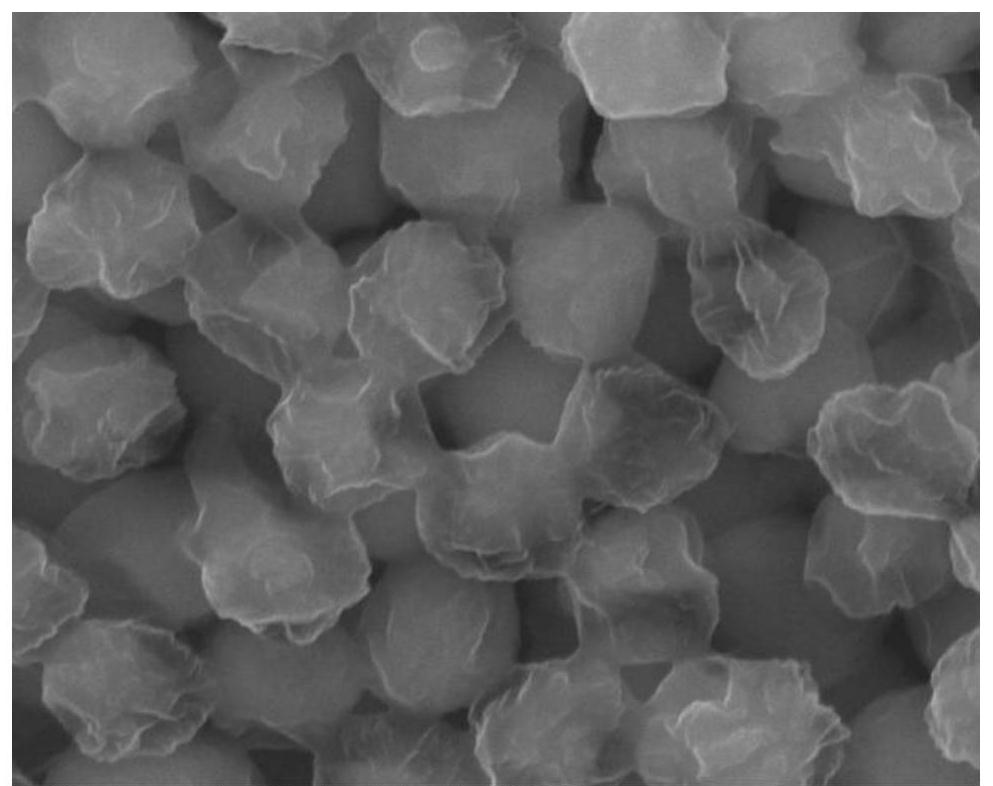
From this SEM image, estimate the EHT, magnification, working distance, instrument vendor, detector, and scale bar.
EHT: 25 kV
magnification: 80 K X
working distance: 11.2 mm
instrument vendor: FEI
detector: ETD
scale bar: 1000 nm